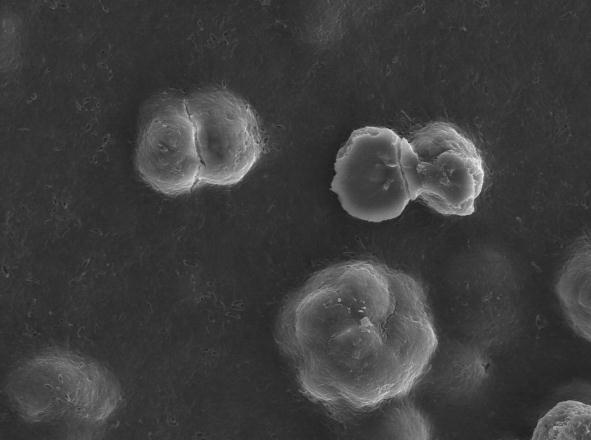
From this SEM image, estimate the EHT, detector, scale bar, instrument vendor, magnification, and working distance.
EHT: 20 kV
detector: SEI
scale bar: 10000 nm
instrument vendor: JEOL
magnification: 1 K X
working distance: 14.9 mm